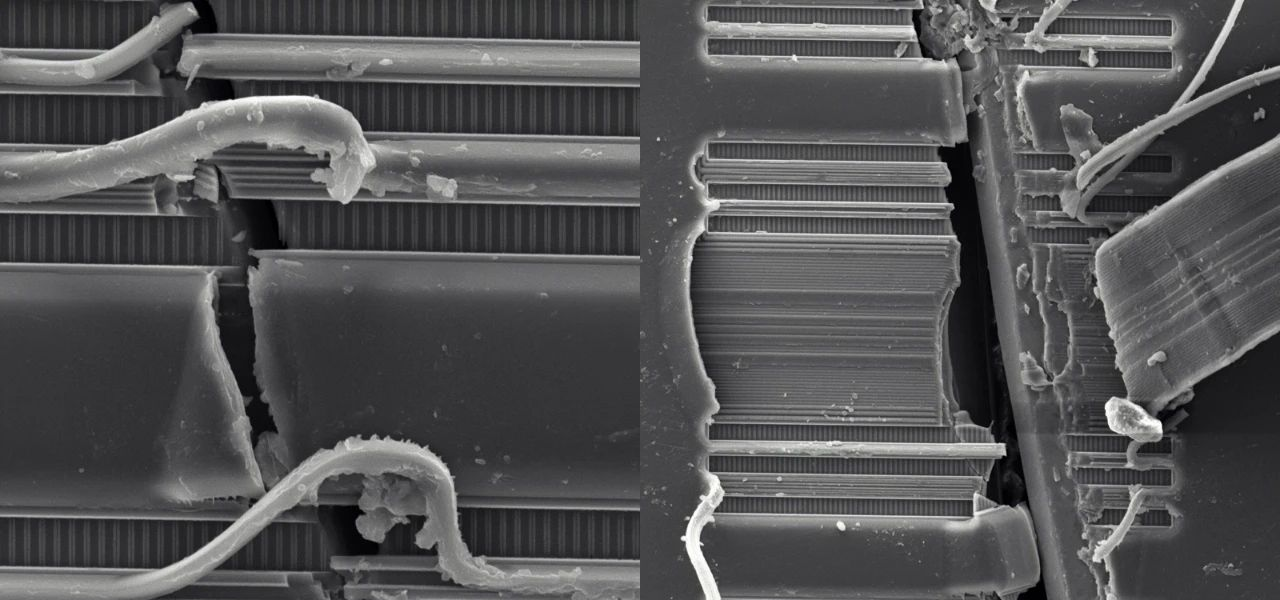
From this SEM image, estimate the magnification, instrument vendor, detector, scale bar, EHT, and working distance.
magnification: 3 K X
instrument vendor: other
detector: SE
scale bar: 10000 nm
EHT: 15 kV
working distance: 6 mm